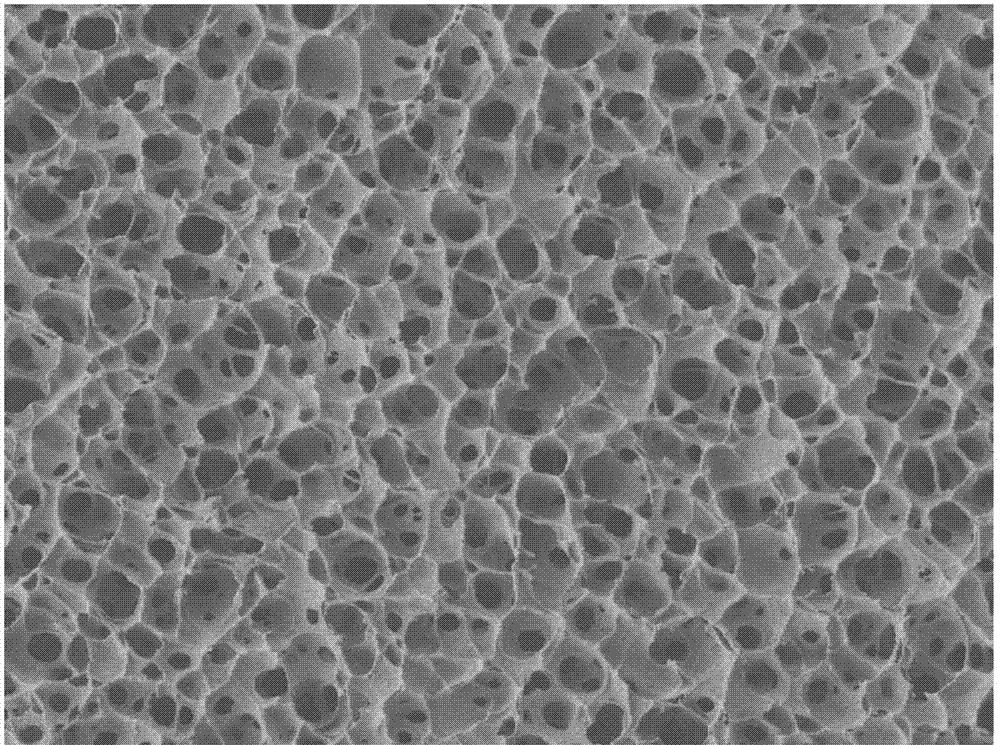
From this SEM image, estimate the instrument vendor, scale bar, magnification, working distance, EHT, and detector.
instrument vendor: JEOL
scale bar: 1000 nm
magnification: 3 K X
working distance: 7.7 mm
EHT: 1 kV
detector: LEI